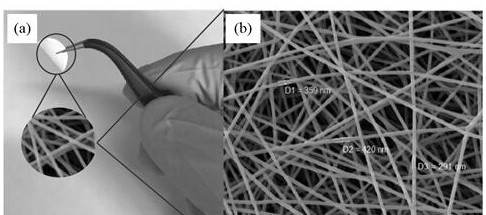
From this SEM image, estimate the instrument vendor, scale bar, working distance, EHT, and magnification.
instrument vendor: Tescan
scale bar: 5000 nm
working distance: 16.42 mm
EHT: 10 kV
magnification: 5 K X